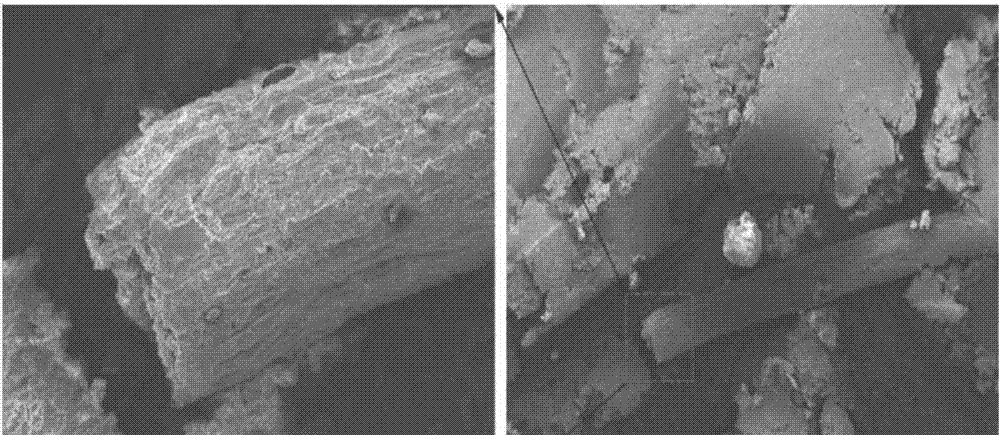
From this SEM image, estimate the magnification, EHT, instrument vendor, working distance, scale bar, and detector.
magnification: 0.135 K X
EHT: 10 kV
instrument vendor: Zeiss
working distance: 16 mm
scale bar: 100000 nm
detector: SE1